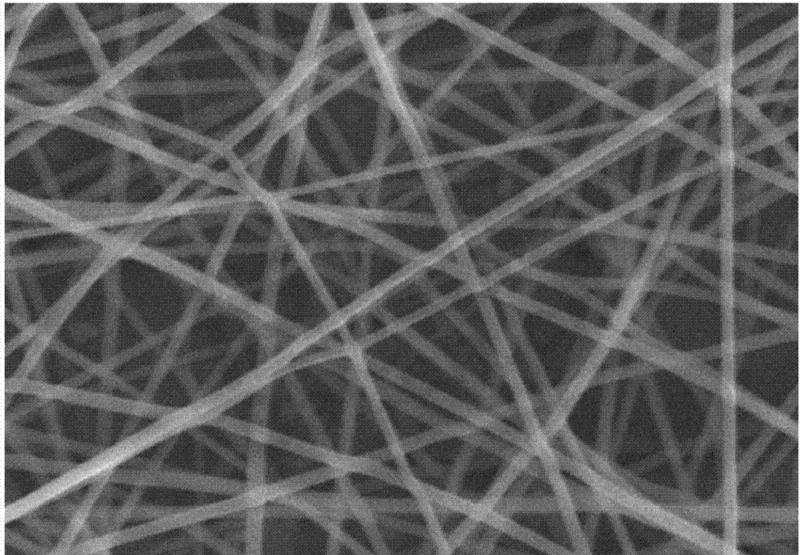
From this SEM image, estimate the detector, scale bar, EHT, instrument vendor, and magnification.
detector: SE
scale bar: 5000 nm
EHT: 10 kV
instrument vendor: Hitachi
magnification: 10 K X